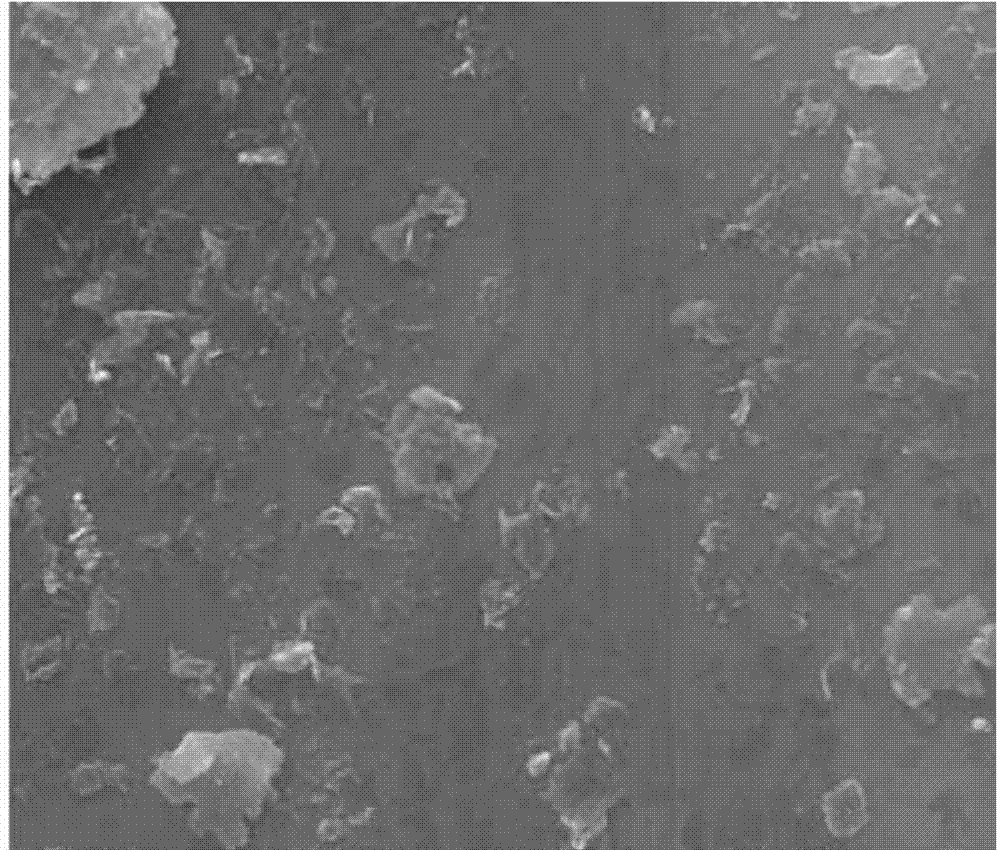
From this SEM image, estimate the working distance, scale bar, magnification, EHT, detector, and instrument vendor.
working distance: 10.2 mm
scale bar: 10000 nm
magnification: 10 K X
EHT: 30 kV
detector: SE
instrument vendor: FEI